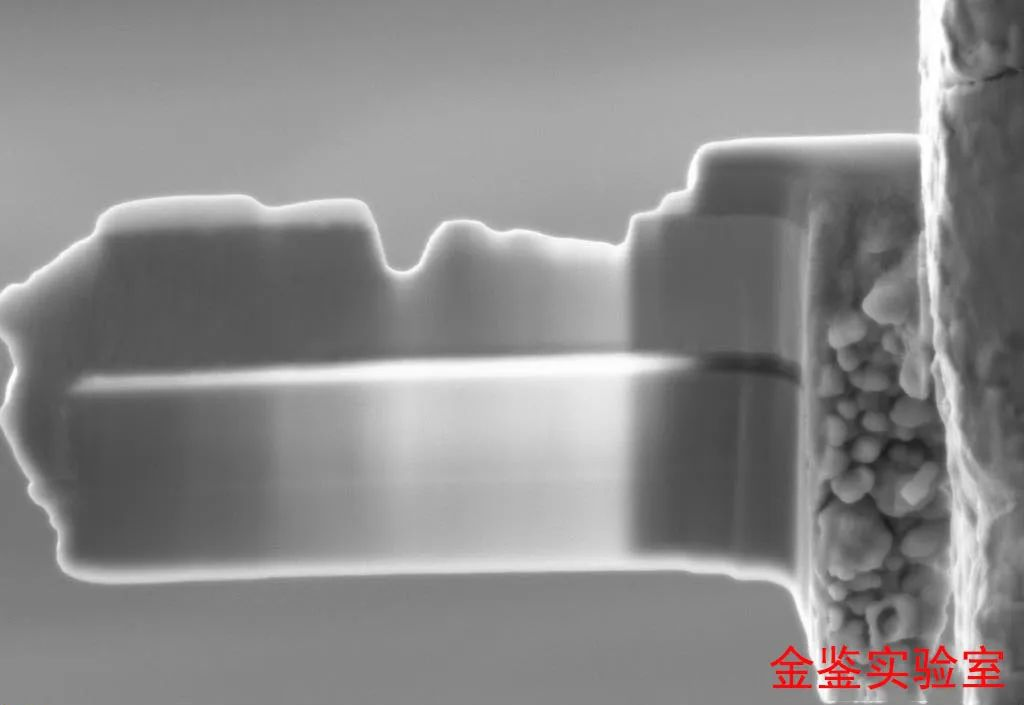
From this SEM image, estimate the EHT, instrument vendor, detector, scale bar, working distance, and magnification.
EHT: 5 kV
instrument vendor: Zeiss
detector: SE2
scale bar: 200 nm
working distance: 5.2 mm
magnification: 17.62 K X